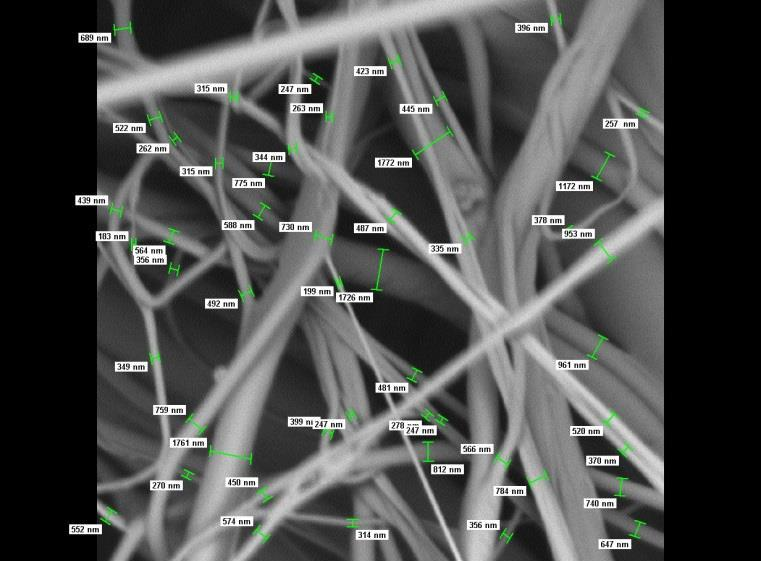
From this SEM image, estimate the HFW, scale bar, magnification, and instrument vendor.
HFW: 24 µm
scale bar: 10000 nm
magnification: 10 K X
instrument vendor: other